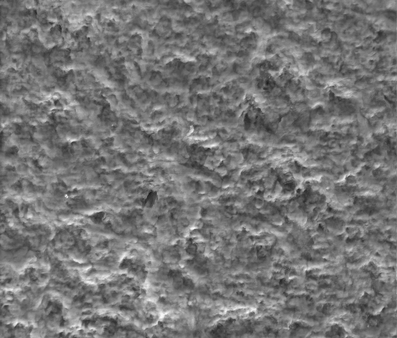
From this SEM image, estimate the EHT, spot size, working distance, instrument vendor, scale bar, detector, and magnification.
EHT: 20 kV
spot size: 4.5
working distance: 8.3 mm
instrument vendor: FEI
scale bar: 50000 nm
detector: GSED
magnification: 1 K X